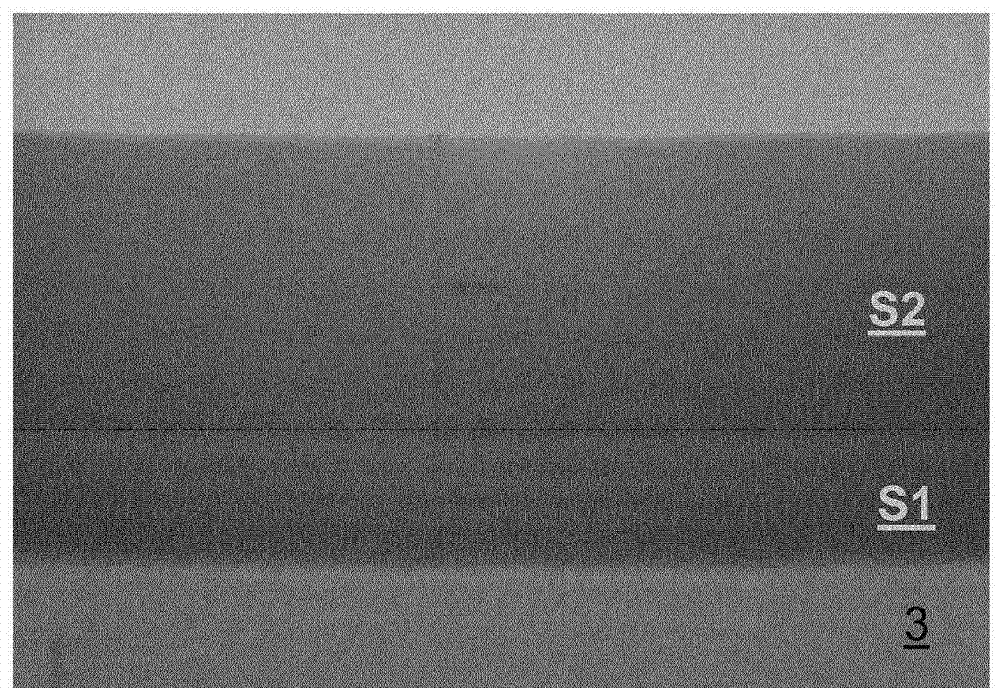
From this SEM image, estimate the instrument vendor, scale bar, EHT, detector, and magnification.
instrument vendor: Hitachi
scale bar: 60 nm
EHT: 200 kV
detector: TE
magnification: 450 K X